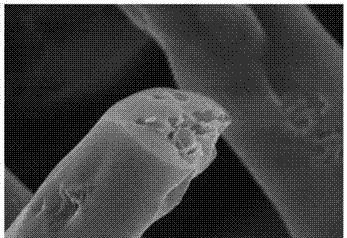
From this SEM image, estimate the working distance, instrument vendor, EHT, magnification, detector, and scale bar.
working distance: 9.2 mm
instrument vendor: Hitachi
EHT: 5 kV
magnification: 50 K X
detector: SE(M)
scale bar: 1000 nm